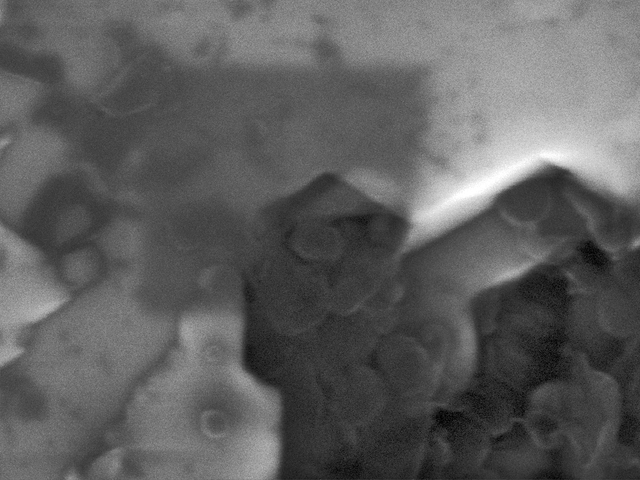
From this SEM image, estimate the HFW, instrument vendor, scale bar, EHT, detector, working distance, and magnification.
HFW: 1.38 µm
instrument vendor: Tescan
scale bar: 200 nm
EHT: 15 kV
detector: In-Beam SE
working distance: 5 mm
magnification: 200 K X